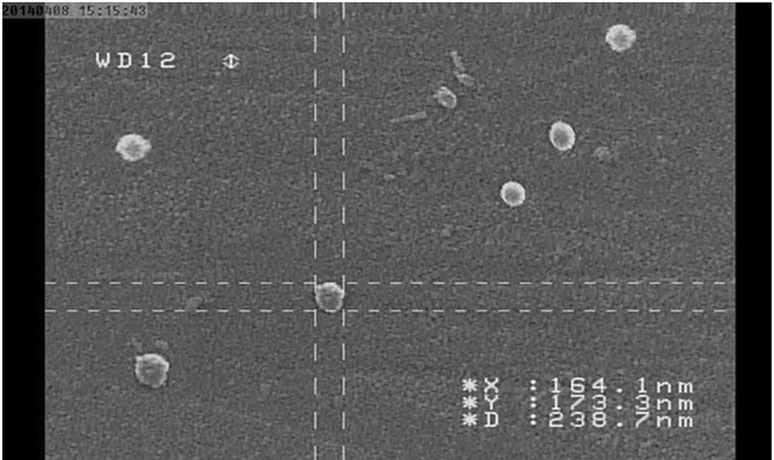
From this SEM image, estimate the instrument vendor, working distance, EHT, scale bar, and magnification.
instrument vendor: JEOL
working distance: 12 mm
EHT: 20 kV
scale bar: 1000 nm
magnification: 30 K X